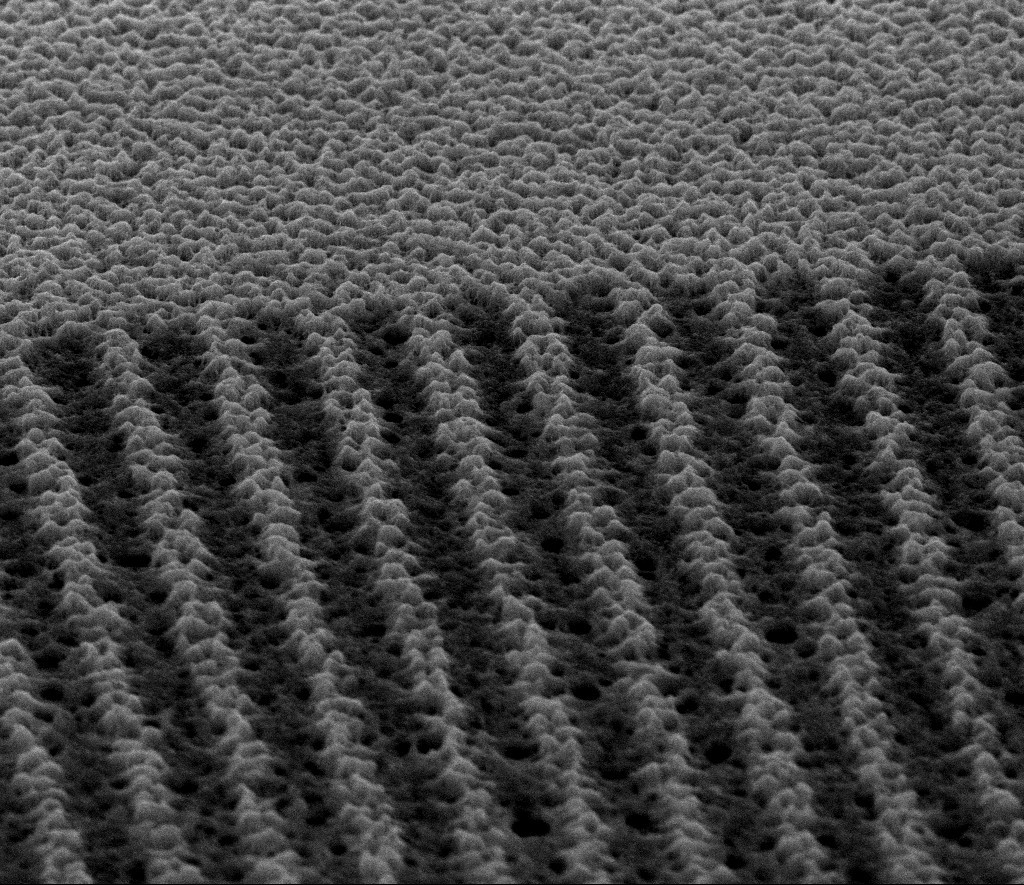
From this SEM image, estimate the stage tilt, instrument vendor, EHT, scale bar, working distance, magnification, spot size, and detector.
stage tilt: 60°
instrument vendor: FEI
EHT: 5 kV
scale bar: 5000 nm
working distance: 10.2 mm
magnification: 20 K X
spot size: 2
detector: ETD